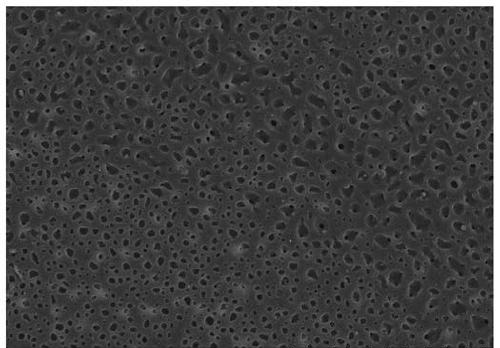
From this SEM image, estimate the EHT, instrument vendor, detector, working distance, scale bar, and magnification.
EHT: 15 kV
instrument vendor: Hitachi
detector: SE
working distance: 4.4 mm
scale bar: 100000 nm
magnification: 0.5 K X